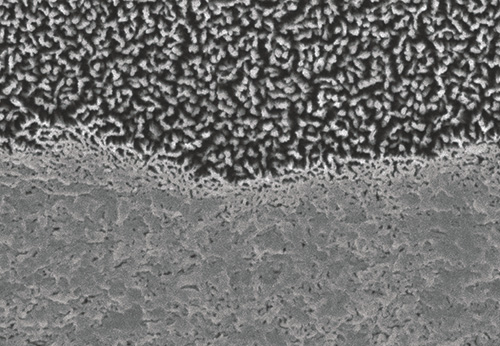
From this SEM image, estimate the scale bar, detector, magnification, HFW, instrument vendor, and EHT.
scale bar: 1000 nm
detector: A+B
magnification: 20 K X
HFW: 6 µm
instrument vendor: Hitachi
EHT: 5 kV